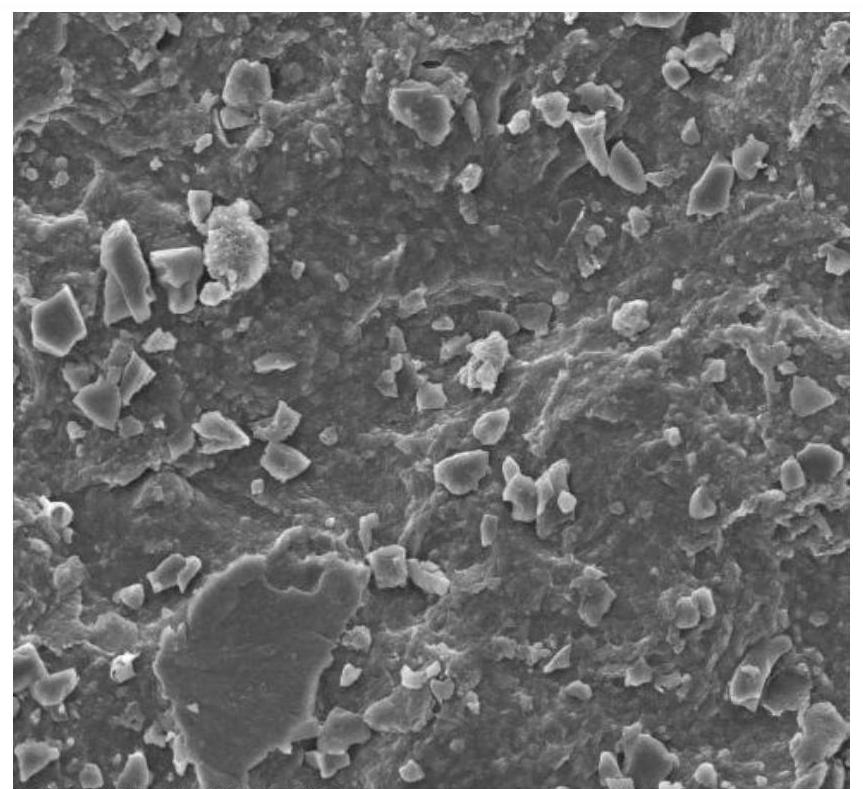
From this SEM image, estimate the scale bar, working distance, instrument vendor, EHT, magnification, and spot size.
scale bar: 100000 nm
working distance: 11 mm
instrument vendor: FEI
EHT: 20 kV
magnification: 0.8 K X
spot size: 3